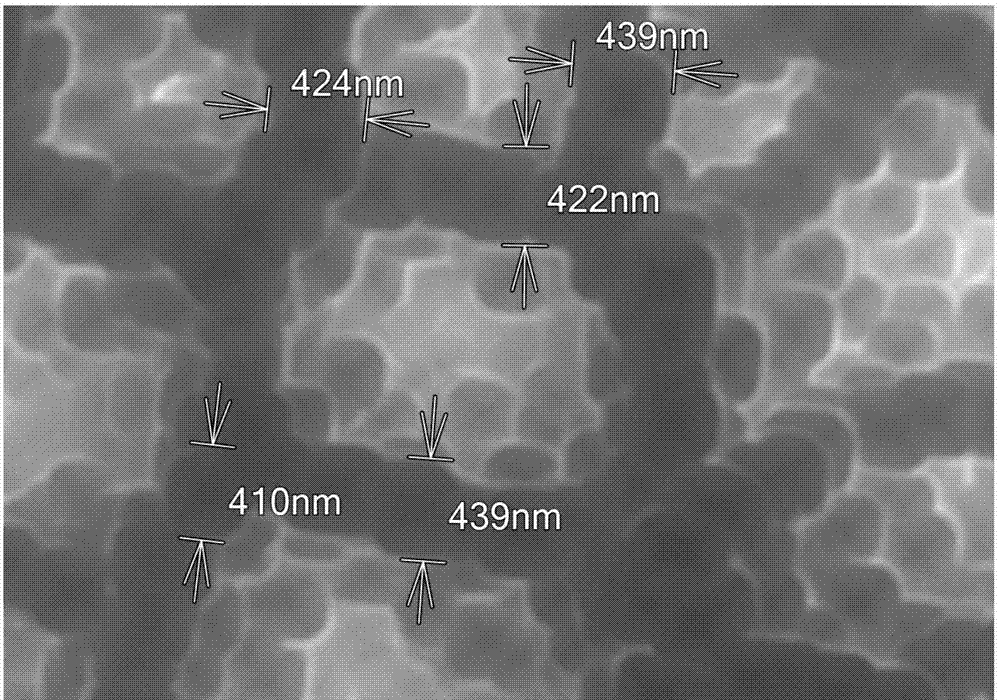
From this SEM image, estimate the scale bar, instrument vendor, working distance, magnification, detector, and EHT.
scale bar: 1000 nm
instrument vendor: Hitachi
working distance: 5.9 mm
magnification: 30 K X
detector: SE(L)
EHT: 20 kV